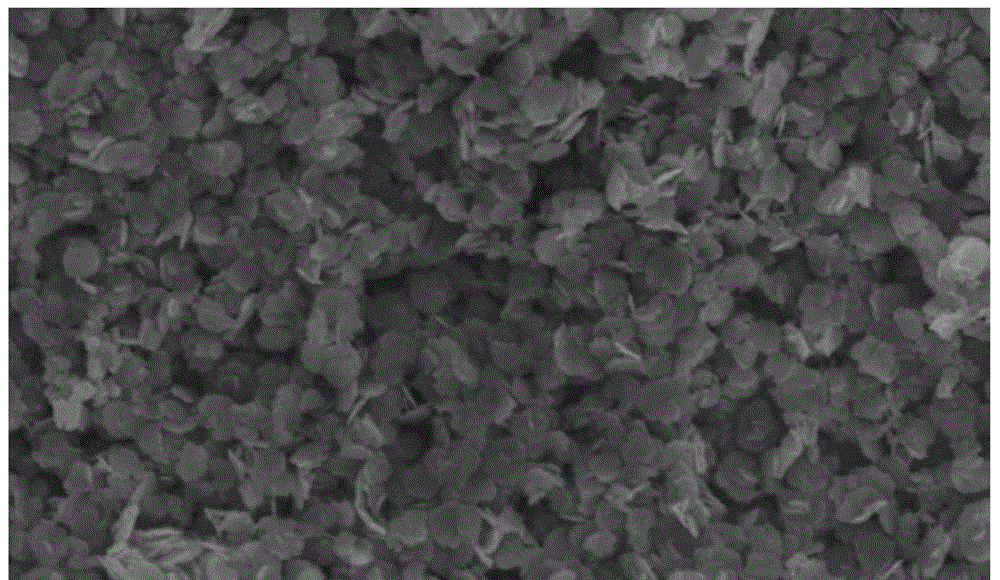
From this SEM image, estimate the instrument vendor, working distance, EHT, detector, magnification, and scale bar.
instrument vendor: Hitachi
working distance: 9.9 mm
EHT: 5 kV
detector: SE(M)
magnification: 20 K X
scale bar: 2000 nm